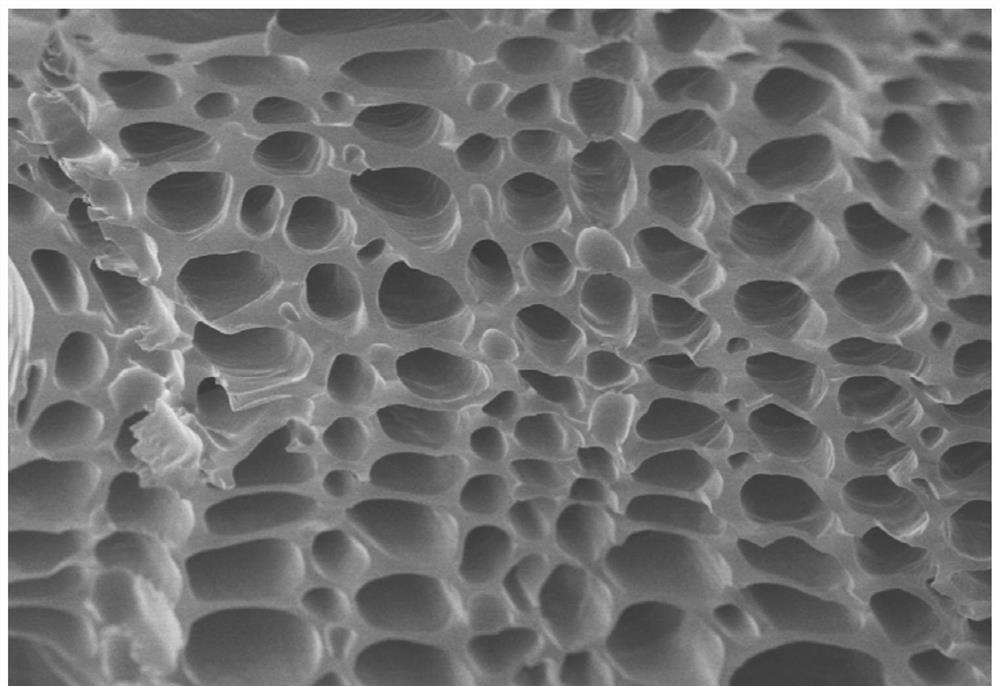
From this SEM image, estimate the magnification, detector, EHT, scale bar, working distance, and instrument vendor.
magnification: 1 K X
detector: SE(UL)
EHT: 5 kV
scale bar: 50000 nm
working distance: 10.2 mm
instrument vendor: Hitachi